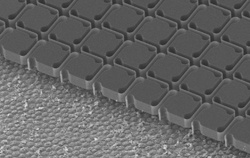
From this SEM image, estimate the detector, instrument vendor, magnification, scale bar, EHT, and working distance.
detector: SE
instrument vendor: Hitachi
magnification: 0.2 K X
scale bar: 200000 nm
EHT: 10 kV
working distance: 38.6 mm